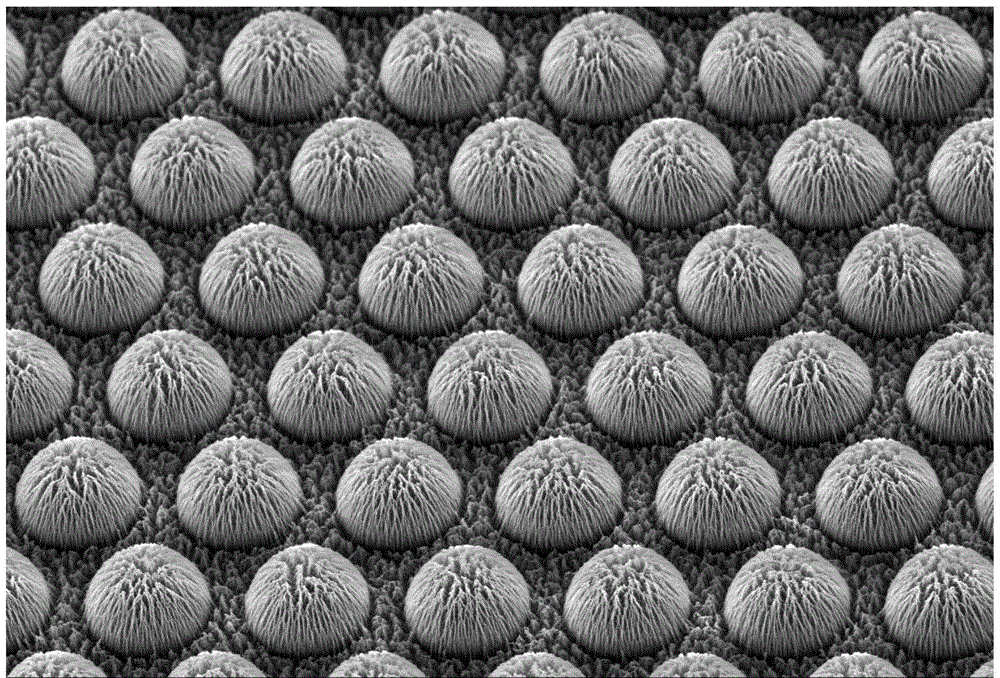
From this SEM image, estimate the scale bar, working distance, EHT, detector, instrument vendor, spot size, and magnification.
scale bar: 20000 nm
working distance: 15 mm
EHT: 20 kV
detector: SEI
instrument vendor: JEOL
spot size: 50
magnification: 0.7 K X